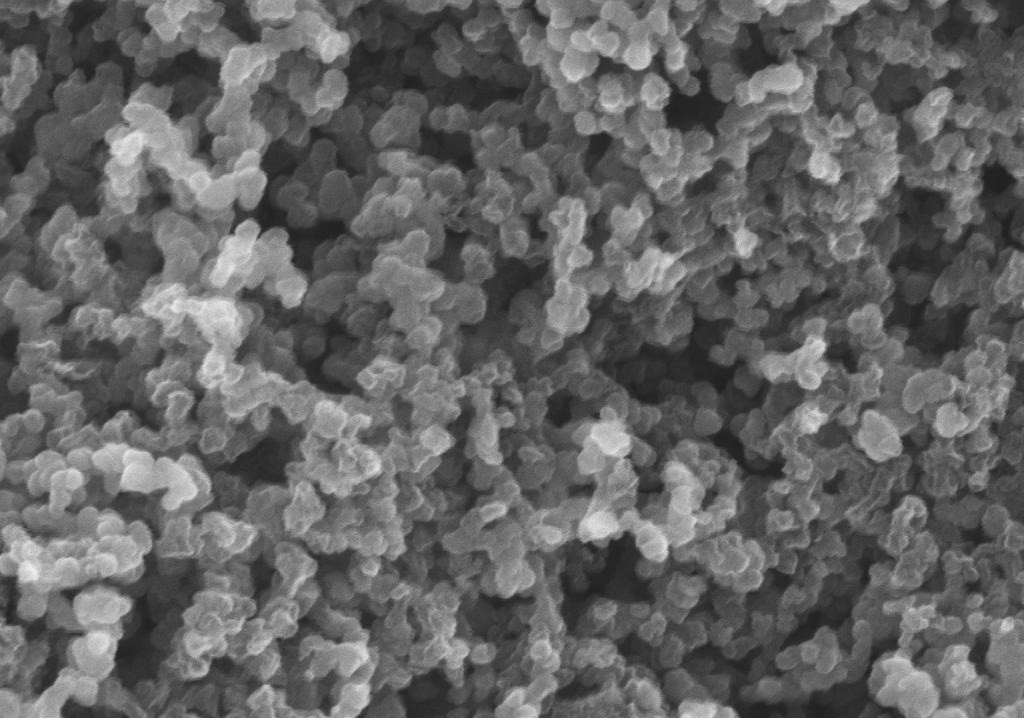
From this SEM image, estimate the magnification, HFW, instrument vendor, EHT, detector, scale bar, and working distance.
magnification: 50 K X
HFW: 2.287 µm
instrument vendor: Zeiss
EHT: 3 kV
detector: InLens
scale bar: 100 nm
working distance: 3.6 mm